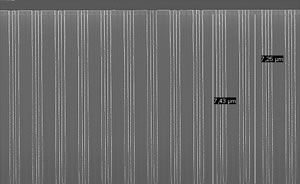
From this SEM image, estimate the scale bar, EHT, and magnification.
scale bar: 3000 nm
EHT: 10 kV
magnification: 11 K X